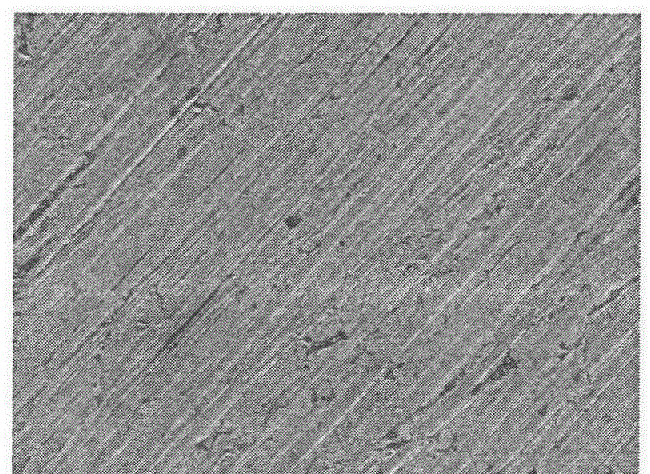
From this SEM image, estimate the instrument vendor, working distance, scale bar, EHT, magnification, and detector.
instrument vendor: JEOL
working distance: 7.7 mm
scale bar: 10000 nm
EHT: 5 kV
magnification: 1.1 K X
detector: LED